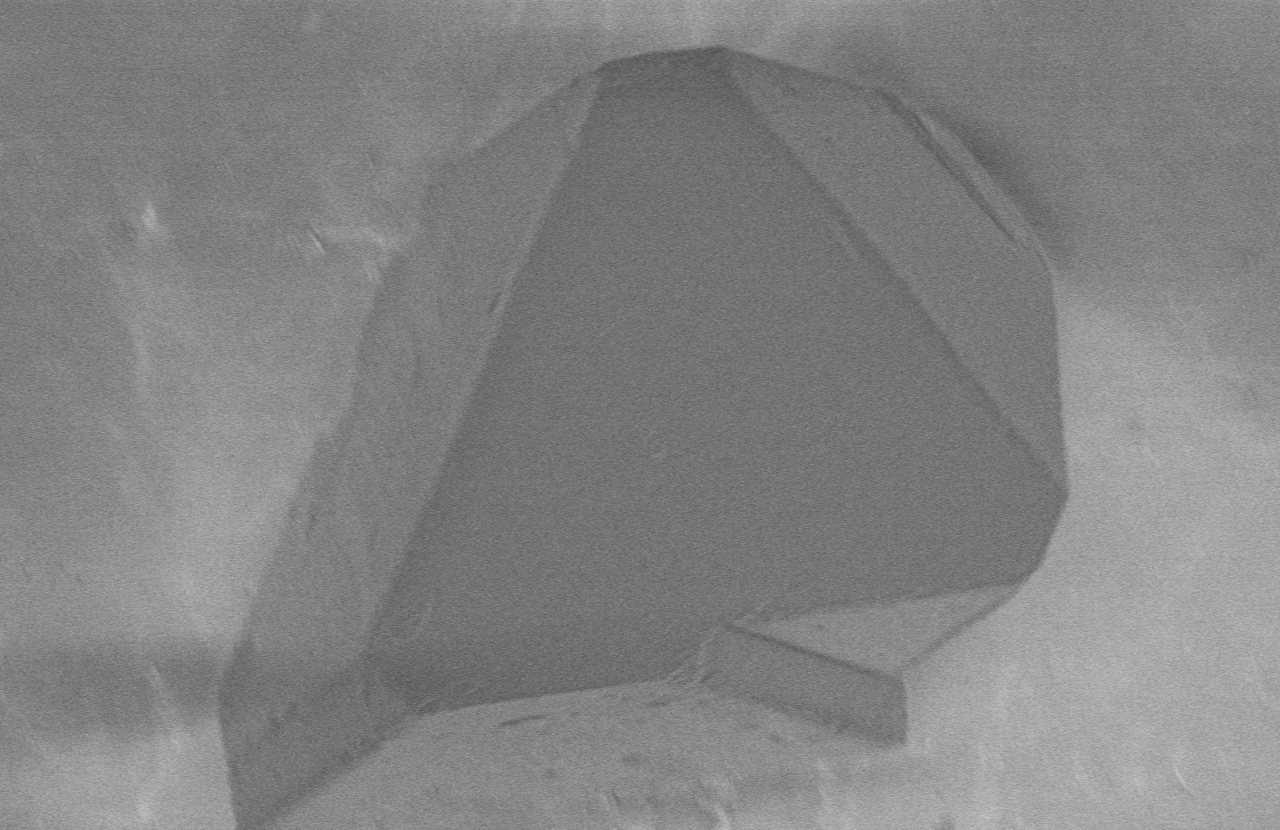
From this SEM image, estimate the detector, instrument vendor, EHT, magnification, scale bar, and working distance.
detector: D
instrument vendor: Hitachi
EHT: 1 kV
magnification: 2.5 K X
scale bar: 20000 nm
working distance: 4.1 mm